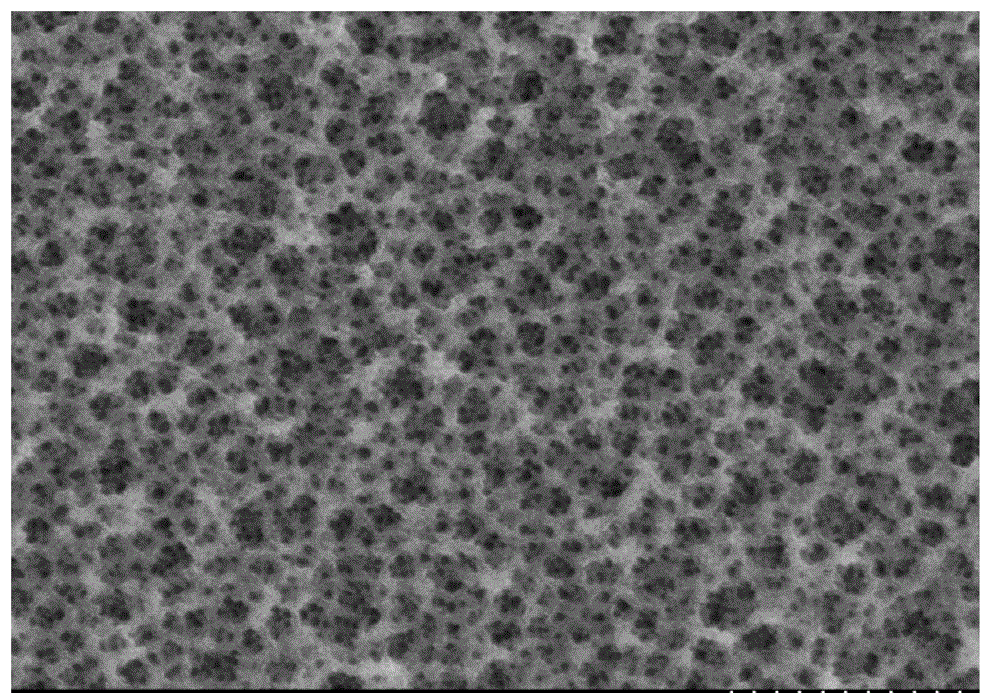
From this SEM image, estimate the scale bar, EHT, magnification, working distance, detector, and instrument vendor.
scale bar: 500 nm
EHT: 5 kV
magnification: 60 K X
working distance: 10.4 mm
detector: SE(M)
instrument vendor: Hitachi